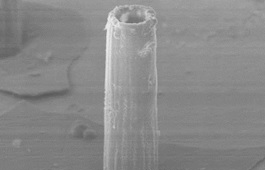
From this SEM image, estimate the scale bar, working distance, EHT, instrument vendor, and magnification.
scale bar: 100000 nm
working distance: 21 mm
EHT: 20 kV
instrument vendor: JEOL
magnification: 0.2 K X